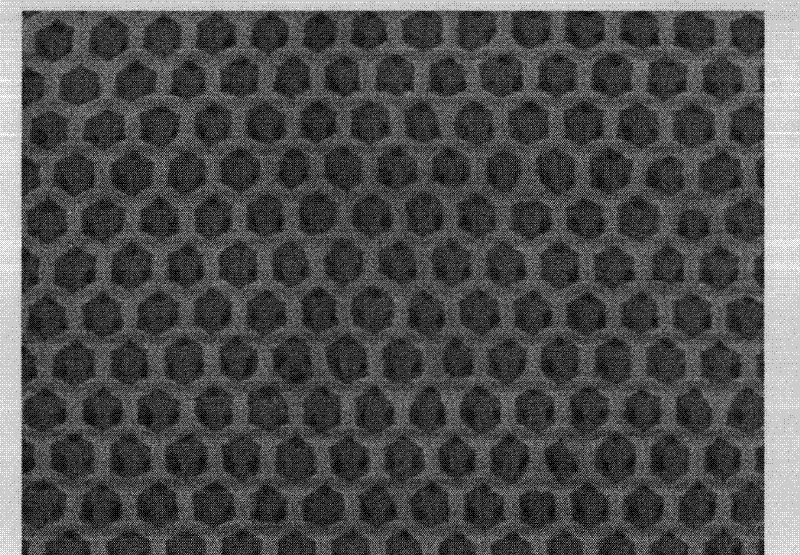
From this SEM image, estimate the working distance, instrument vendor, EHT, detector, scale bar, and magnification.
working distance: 6.6 mm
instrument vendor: JEOL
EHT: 5 kV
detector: SEI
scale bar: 100 nm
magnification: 43 K X